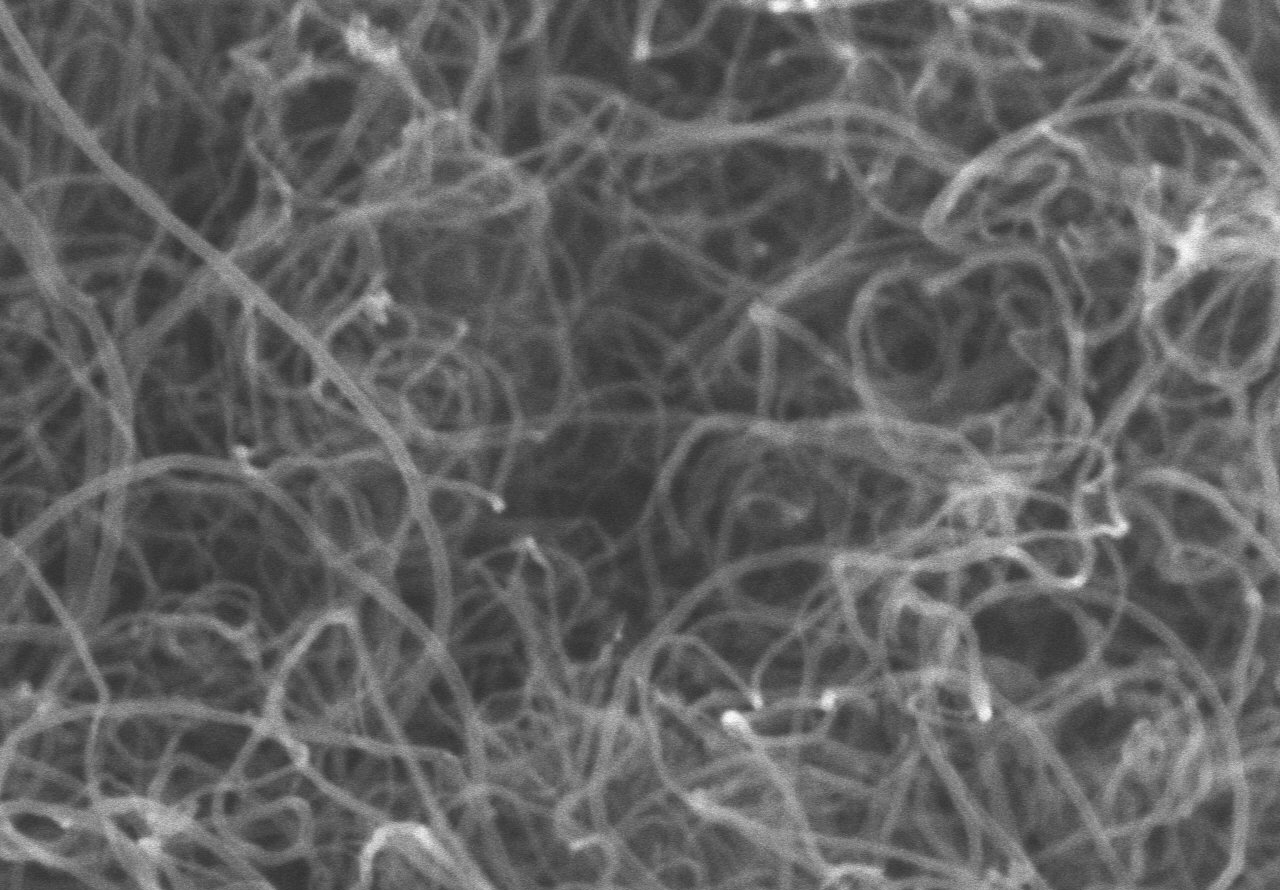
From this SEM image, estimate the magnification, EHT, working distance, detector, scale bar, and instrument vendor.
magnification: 80 K X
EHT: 5 kV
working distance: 4.9 mm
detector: SE(M)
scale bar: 500 nm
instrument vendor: Hitachi